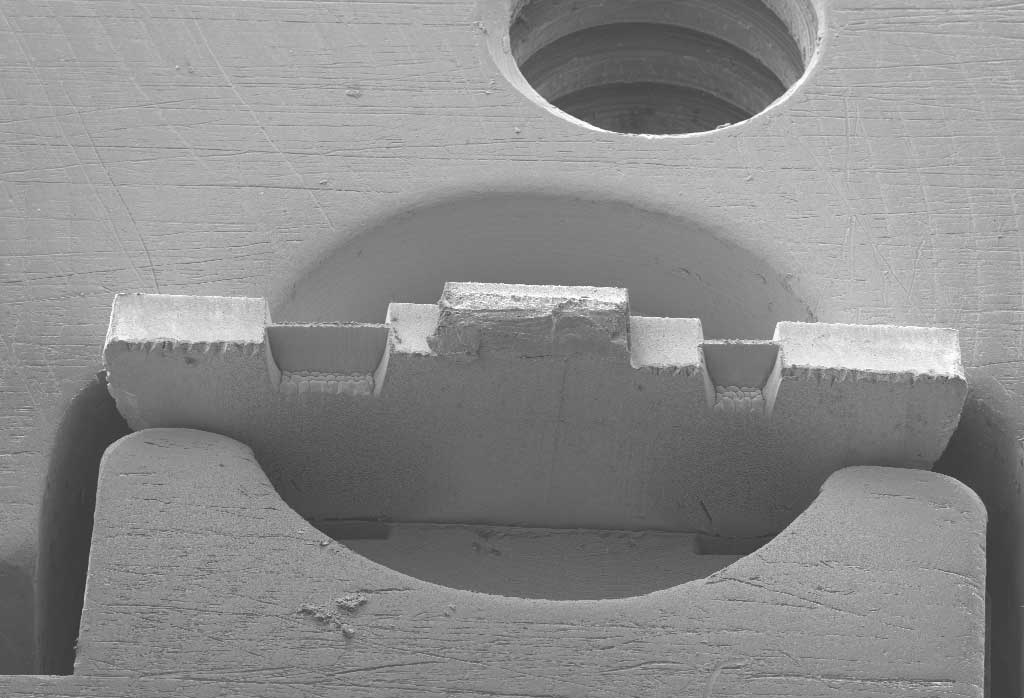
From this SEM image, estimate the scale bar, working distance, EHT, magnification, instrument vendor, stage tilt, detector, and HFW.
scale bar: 200000 nm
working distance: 10.5 mm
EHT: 10 kV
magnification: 0.035 K X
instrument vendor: Zeiss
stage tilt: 45°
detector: SESI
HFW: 3267 µm